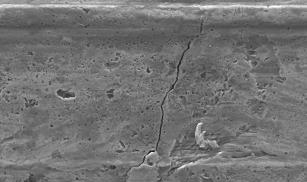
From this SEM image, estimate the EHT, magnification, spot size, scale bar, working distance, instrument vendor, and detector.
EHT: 20 kV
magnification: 1 K X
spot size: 4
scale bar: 20000 nm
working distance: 11.1 mm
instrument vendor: FEI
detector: SE